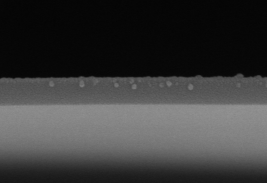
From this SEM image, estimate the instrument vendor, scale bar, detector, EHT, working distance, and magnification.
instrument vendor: Hitachi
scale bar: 500 nm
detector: SE(M)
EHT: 10 kV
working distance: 8.5 mm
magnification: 90.1 K X